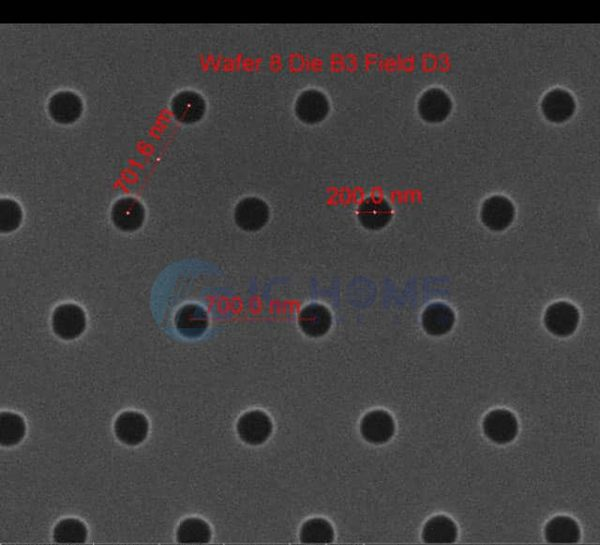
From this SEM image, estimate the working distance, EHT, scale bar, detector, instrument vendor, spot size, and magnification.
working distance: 10.4 mm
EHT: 10 kV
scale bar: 1000 nm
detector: ETD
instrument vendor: FEI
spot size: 1.5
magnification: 75 K X